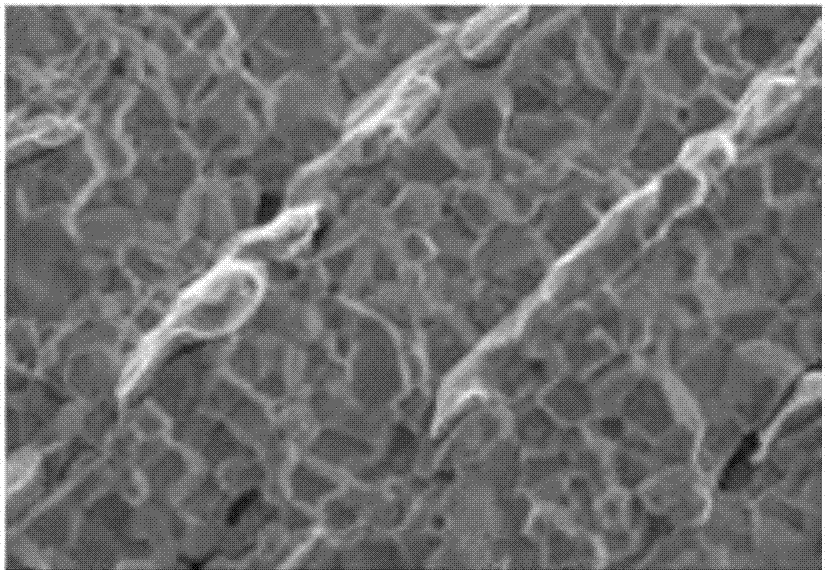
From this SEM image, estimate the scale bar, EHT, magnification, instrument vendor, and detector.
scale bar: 2000 nm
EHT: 5 kV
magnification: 8 K X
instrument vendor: JEOL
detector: SEI